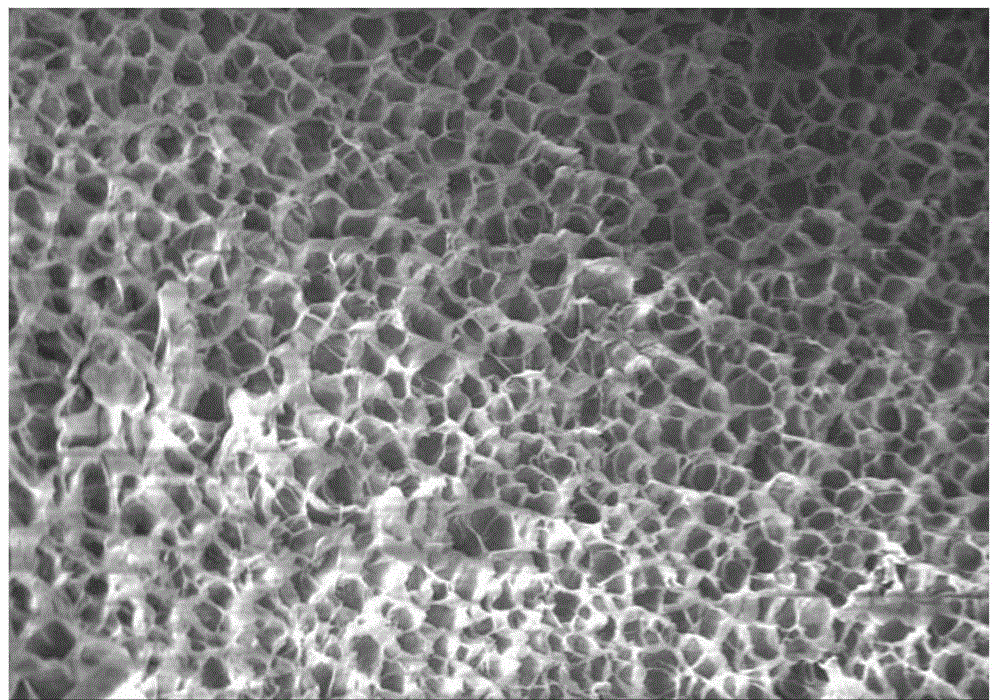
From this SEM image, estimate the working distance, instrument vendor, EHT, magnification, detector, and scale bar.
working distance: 14.4 mm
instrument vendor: Hitachi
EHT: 10 kV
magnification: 1 K X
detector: SE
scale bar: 50000 nm